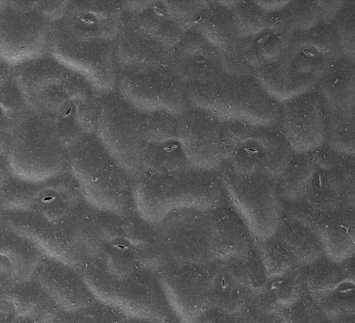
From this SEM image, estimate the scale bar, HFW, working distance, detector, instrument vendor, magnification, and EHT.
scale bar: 50000 nm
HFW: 298 µm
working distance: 10 mm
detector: ETD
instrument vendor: FEI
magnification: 0.5 K X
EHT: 5 kV